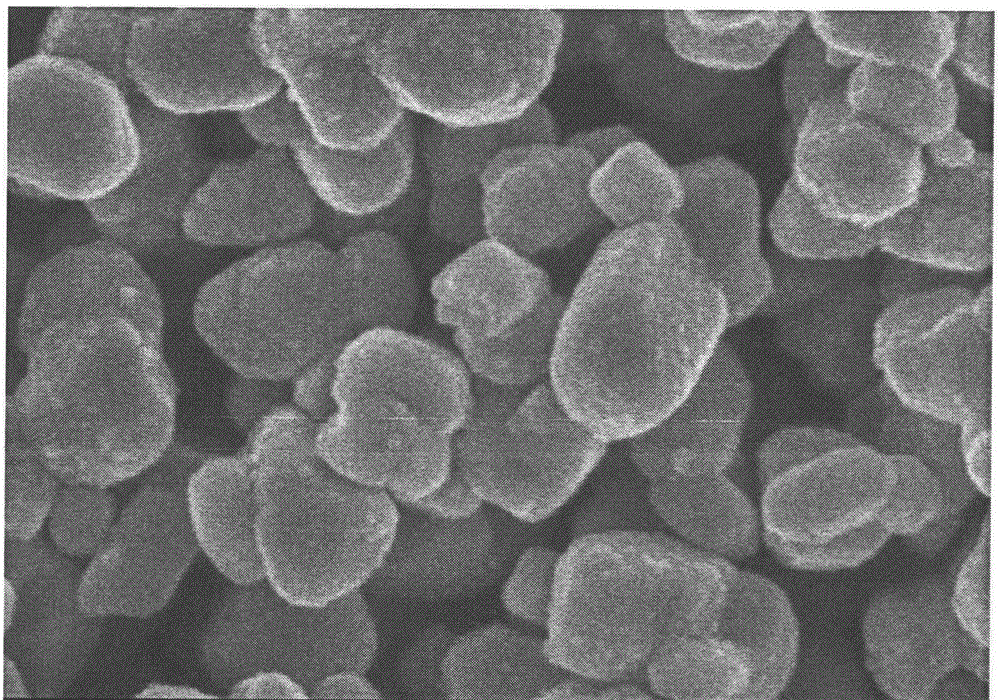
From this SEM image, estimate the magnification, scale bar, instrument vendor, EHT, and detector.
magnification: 80 K X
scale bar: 500 nm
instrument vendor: Hitachi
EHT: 4 kV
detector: SE(U)